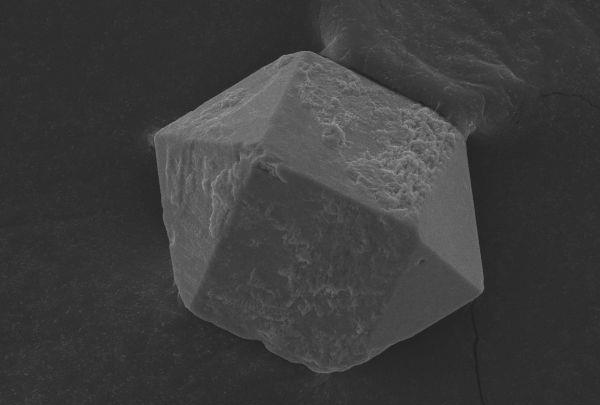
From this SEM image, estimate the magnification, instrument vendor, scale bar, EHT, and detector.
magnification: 0.95 K X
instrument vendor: JEOL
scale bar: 20000 nm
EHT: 20 kV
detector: SEI